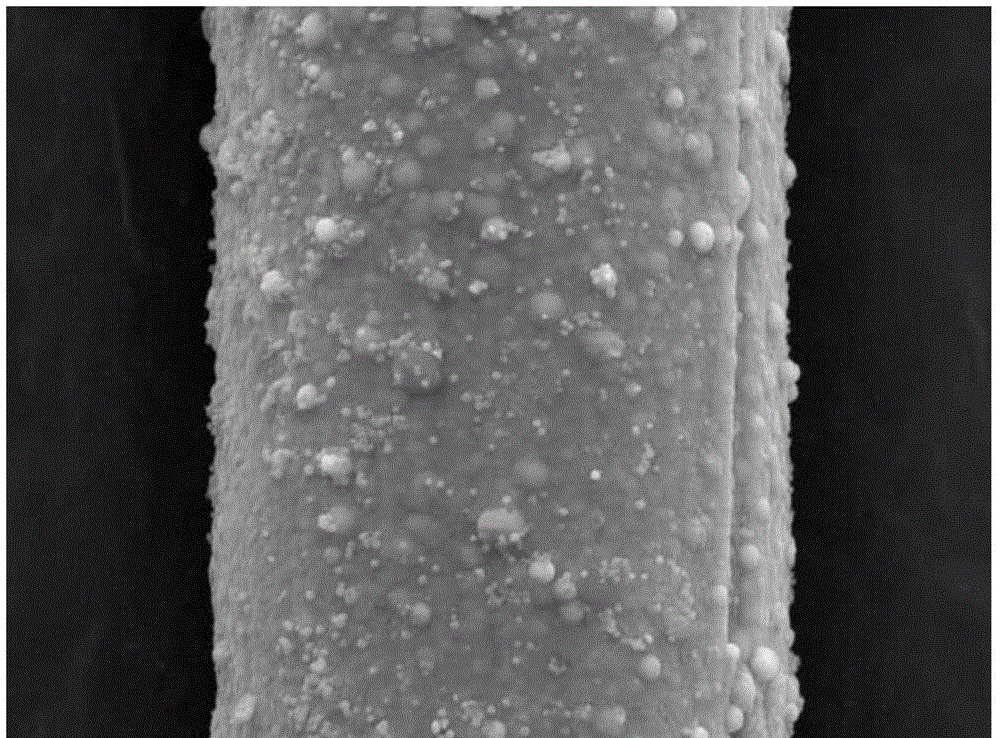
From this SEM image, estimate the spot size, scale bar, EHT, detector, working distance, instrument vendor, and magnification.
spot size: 5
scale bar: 5000 nm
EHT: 20 kV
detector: SE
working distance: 24 mm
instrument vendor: FEI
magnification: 5 K X